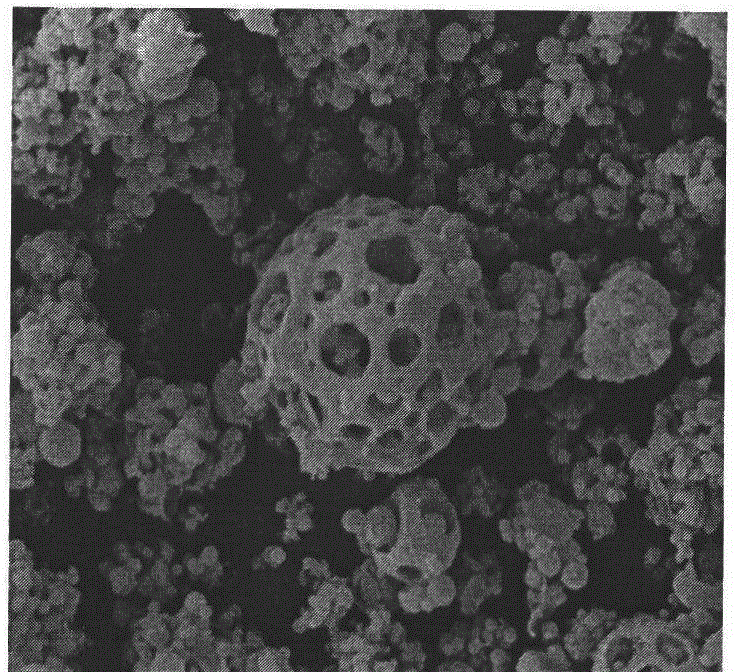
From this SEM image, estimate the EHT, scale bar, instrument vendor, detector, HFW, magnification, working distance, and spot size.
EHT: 20 kV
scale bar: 20000 nm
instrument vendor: FEI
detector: ETD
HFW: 59.7 µm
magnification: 5 K X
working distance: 10.5 mm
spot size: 5